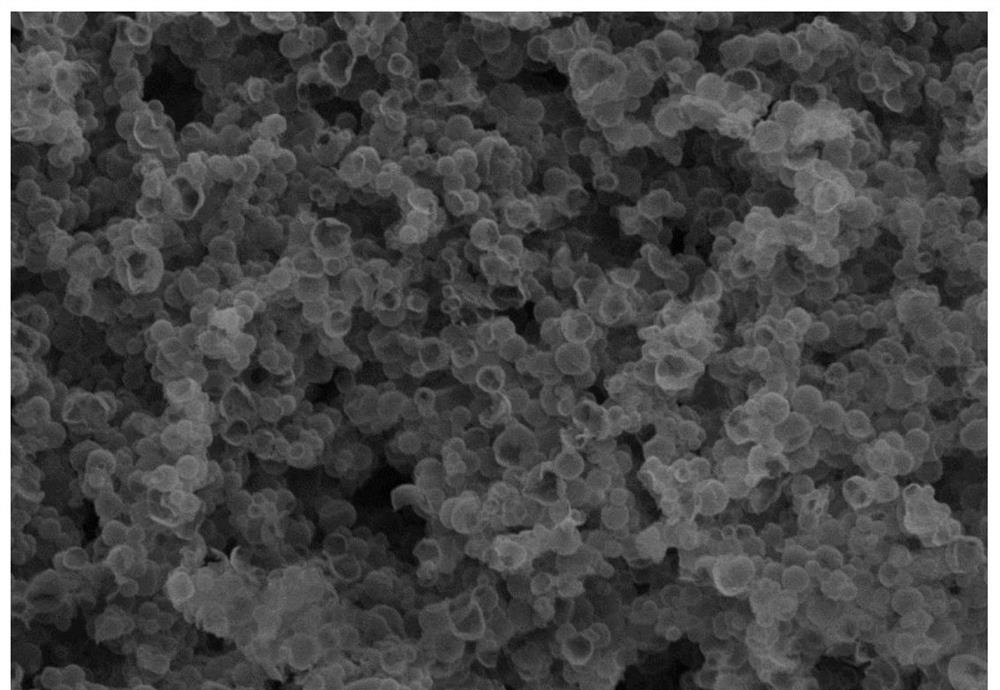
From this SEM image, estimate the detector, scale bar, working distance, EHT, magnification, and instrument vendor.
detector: SE(U)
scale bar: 10000 nm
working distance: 10.4 mm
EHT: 10 kV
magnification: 5 K X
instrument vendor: Hitachi